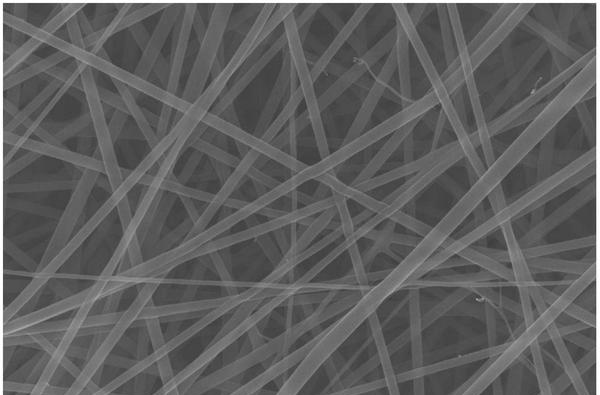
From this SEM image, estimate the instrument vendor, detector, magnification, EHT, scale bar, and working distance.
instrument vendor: Zeiss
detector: InLens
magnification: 20 K X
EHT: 20 kV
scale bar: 200 nm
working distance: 5.8 mm